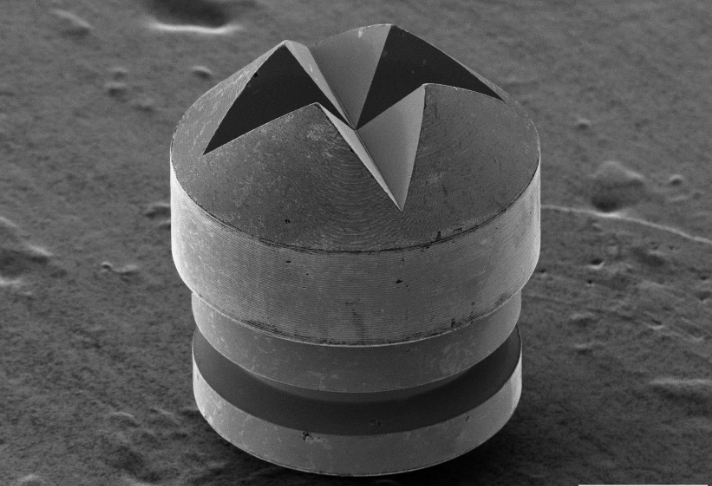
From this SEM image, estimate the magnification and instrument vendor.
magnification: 0.25 K X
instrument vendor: other